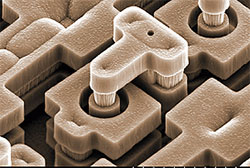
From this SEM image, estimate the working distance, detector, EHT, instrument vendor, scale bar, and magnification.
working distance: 12.5 mm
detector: SE(M)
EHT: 10 kV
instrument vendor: Hitachi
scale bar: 10000 nm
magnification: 5 K X